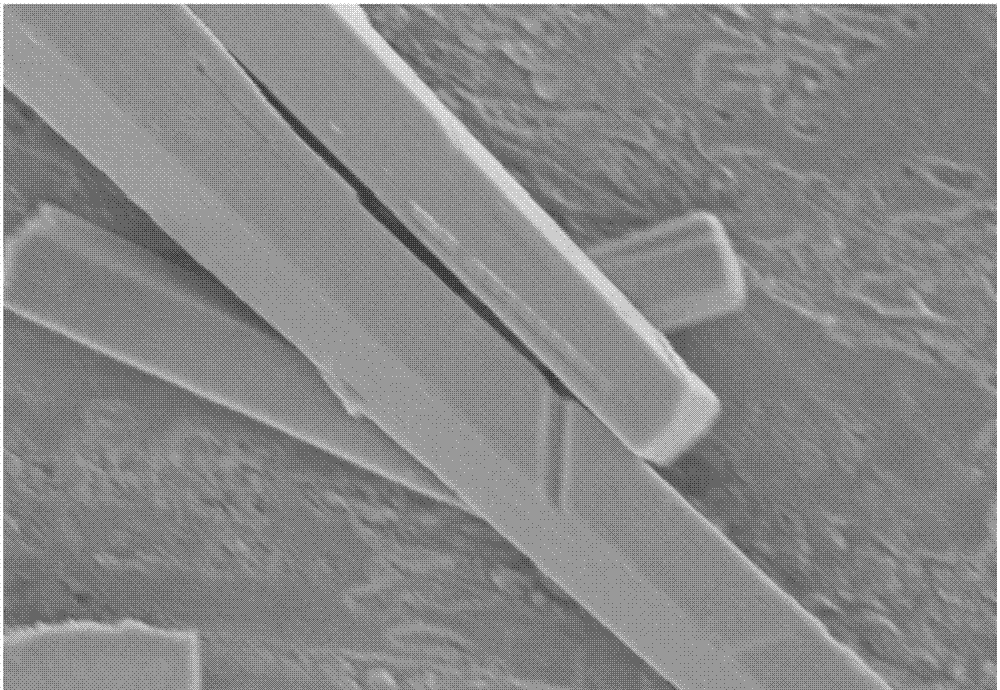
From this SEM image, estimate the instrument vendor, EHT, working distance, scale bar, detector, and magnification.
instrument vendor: Hitachi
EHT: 5 kV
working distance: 9.8 mm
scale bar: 5000 nm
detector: SE(M)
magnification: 7 K X